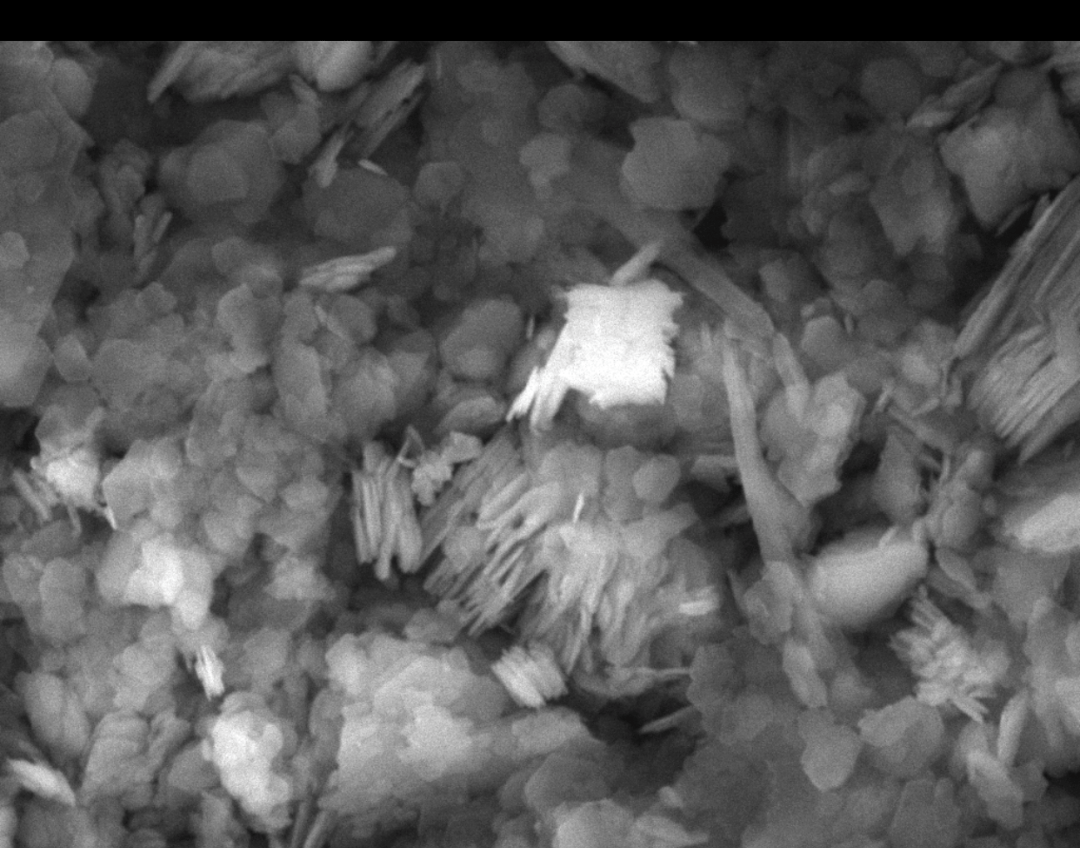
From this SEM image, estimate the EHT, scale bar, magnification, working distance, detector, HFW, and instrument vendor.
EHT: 15 kV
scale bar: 2000 nm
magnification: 20 K X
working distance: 7.74 mm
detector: SE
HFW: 13.8 µm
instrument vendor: Tescan